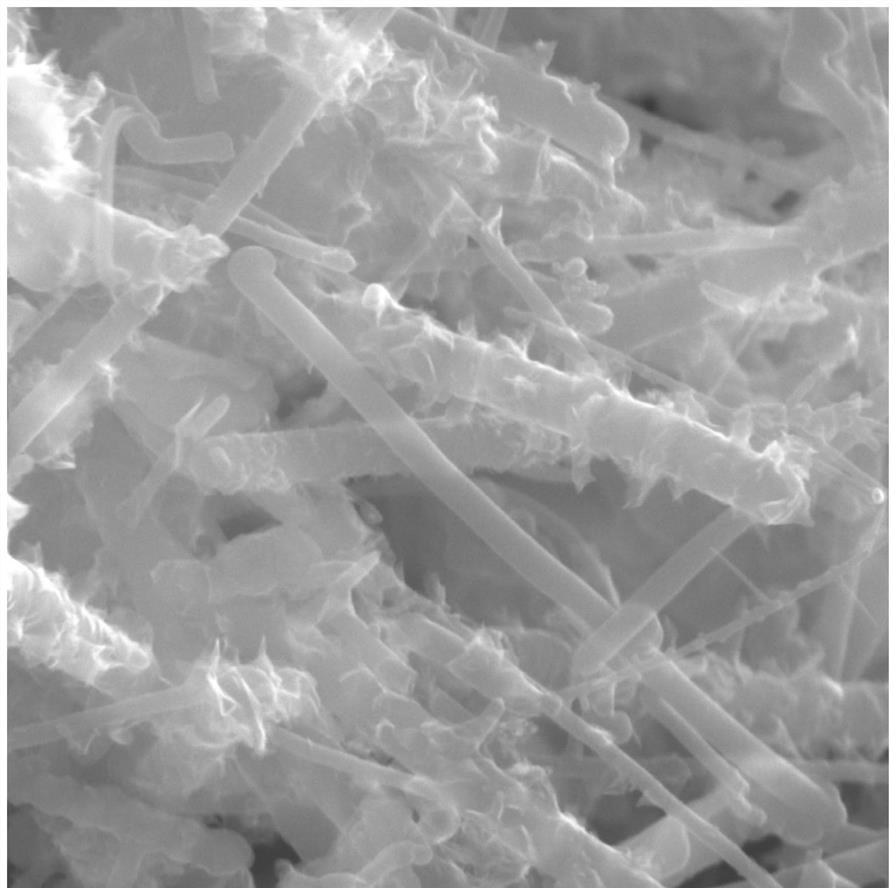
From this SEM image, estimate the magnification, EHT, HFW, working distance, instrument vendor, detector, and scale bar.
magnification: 100 K X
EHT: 15 kV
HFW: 2.08 µm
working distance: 5.01 mm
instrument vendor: Tescan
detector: In-Beam SE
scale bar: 500 nm